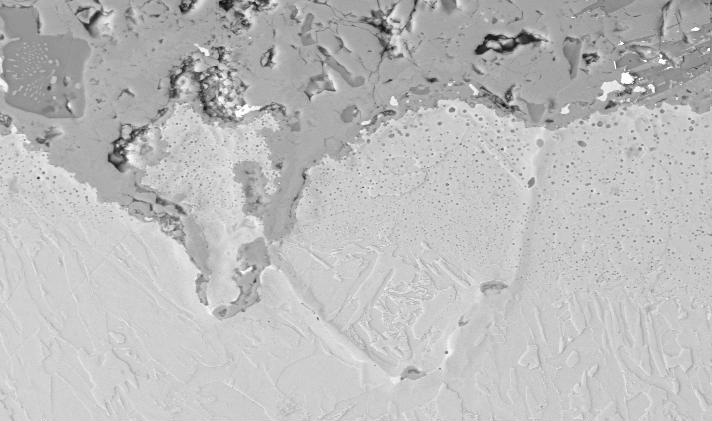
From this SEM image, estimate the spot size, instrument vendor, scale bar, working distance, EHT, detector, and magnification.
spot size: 3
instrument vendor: FEI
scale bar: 20000 nm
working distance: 5.2 mm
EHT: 20 kV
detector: BSE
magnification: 1 K X